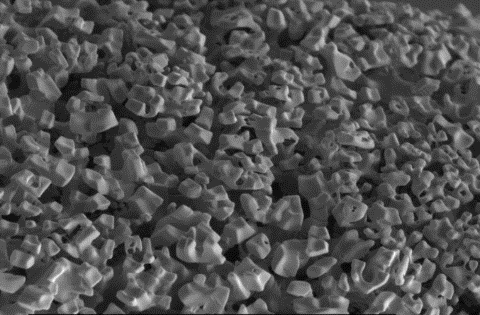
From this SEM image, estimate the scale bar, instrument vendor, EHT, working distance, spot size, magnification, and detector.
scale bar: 20000 nm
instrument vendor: FEI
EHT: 25 kV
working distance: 5.5 mm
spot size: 5.6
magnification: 1.2 K X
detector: SE